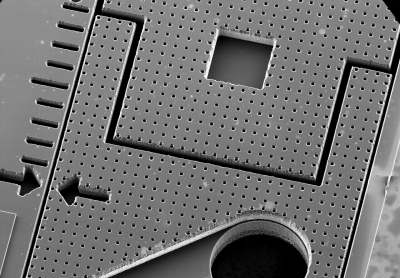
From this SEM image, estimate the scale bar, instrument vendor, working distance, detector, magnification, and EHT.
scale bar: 1000000 nm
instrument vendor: Hitachi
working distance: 5.9 mm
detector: SE(U)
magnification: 0.035 K X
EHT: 25 kV